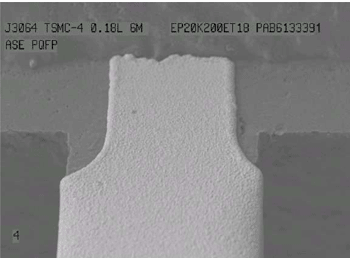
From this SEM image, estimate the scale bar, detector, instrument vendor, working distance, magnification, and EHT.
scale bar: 100000 nm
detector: LEI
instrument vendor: JEOL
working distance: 14 mm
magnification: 0.25 K X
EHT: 15 kV